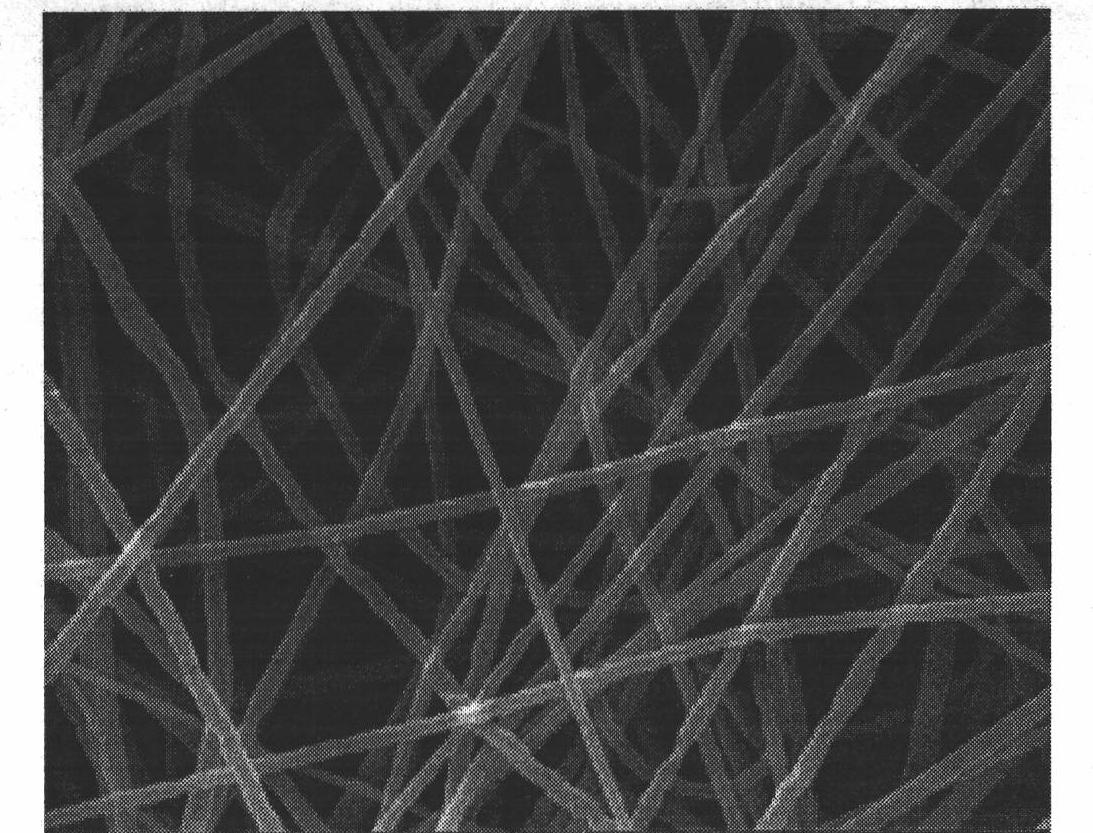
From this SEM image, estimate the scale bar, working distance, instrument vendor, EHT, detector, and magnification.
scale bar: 10000 nm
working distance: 7.9 mm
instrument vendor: FEI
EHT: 30 kV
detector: SE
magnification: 10 K X